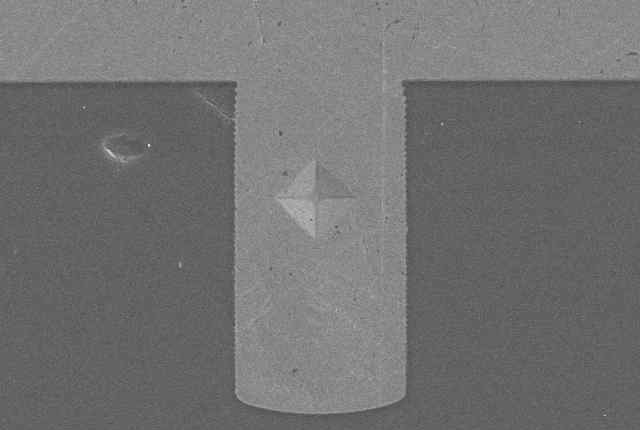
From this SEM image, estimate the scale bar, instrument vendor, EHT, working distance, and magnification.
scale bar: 25000 nm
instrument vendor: Hitachi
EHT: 15 kV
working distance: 14.7 mm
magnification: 1.2 K X